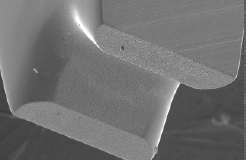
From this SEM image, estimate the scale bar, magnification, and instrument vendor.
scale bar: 100000 nm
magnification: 0.15 K X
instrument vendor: JEOL